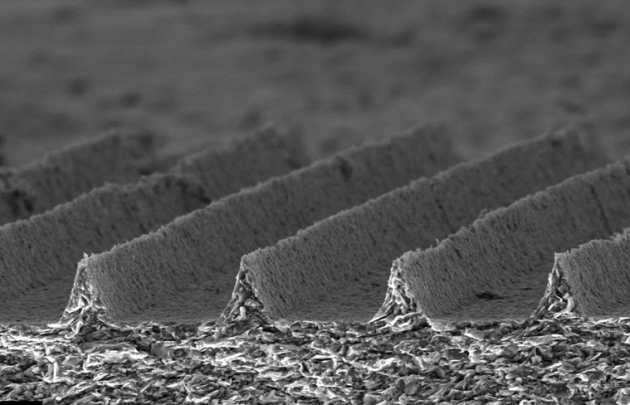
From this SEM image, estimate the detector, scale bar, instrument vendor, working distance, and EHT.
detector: SE1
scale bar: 20000 nm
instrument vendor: Zeiss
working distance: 11 mm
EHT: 20 kV